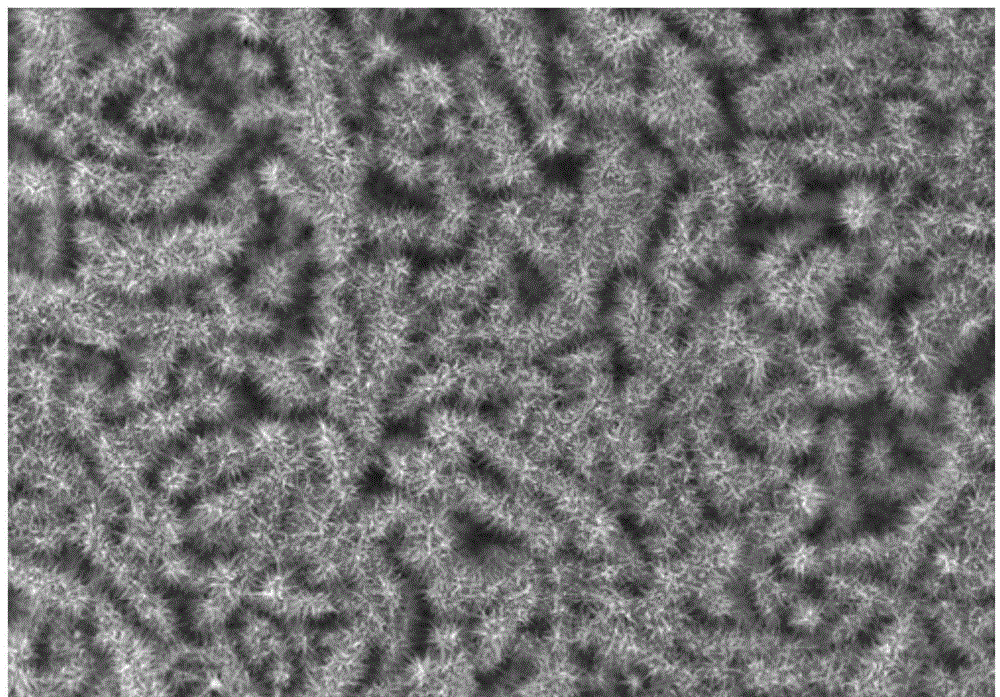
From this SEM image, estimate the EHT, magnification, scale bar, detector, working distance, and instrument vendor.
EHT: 10 kV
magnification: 2 K X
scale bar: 20000 nm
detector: SE(M)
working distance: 5 mm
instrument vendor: Hitachi